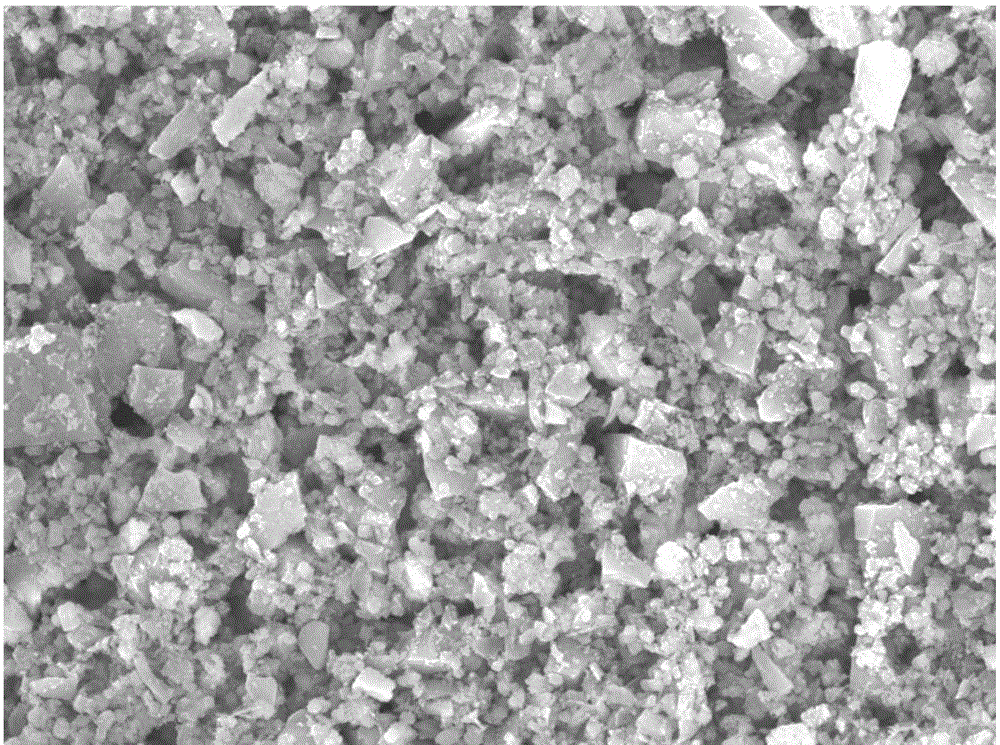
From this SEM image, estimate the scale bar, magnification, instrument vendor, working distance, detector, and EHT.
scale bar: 1000 nm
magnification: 5 K X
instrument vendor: JEOL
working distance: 10.5 mm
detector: SEI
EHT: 15 kV